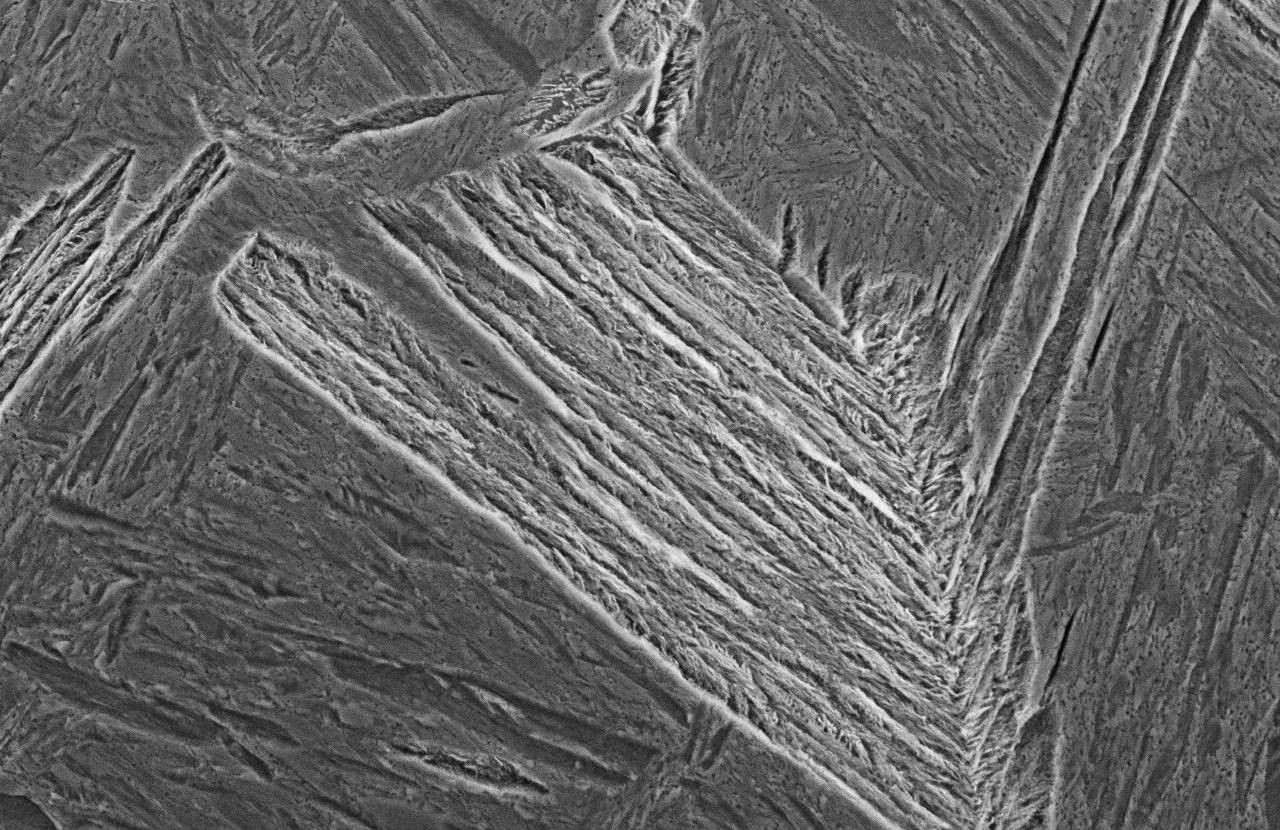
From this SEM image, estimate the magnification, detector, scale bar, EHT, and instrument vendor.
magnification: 2 K X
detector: SEI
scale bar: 10000 nm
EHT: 15 kV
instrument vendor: JEOL